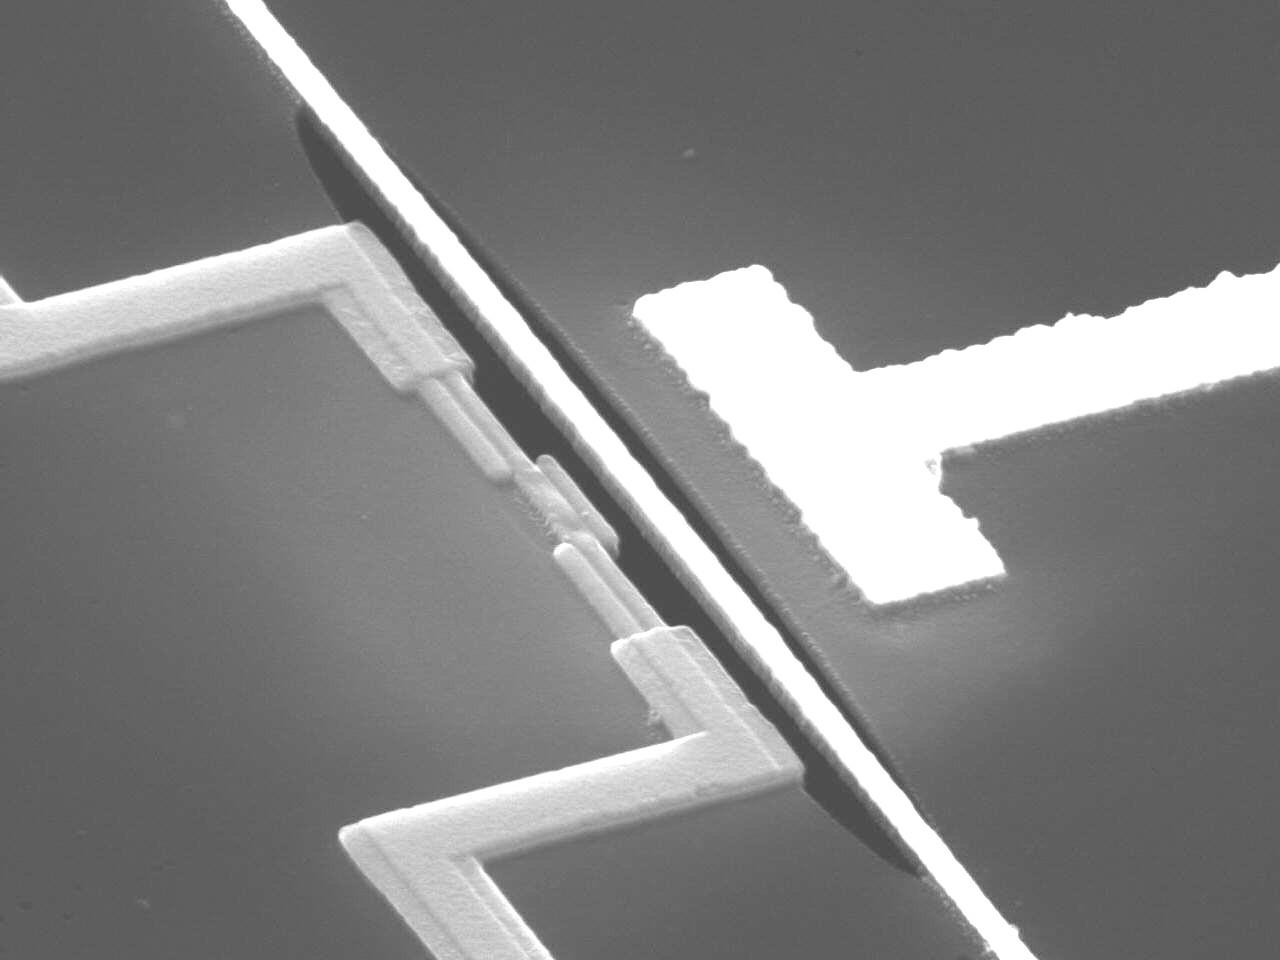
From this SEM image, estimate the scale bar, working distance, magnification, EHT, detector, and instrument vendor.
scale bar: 1000 nm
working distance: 20 mm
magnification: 14 K X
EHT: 15 kV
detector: SEI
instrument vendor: JEOL